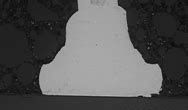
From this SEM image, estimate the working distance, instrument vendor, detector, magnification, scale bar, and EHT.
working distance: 12.5 mm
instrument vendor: Hitachi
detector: BSECOMP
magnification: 0.8 K X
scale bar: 50000 nm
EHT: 10 kV